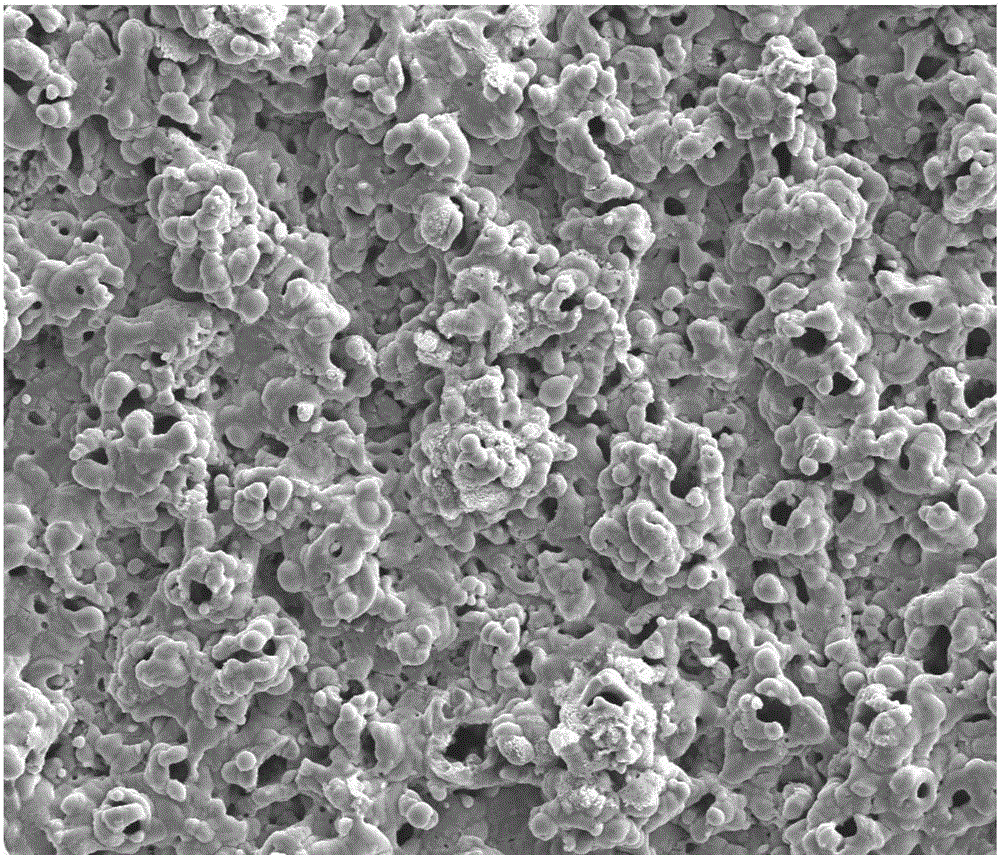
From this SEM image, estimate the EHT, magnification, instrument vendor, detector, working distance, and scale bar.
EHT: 20 kV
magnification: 1 K X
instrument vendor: FEI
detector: SE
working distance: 5.1 mm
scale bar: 50000 nm